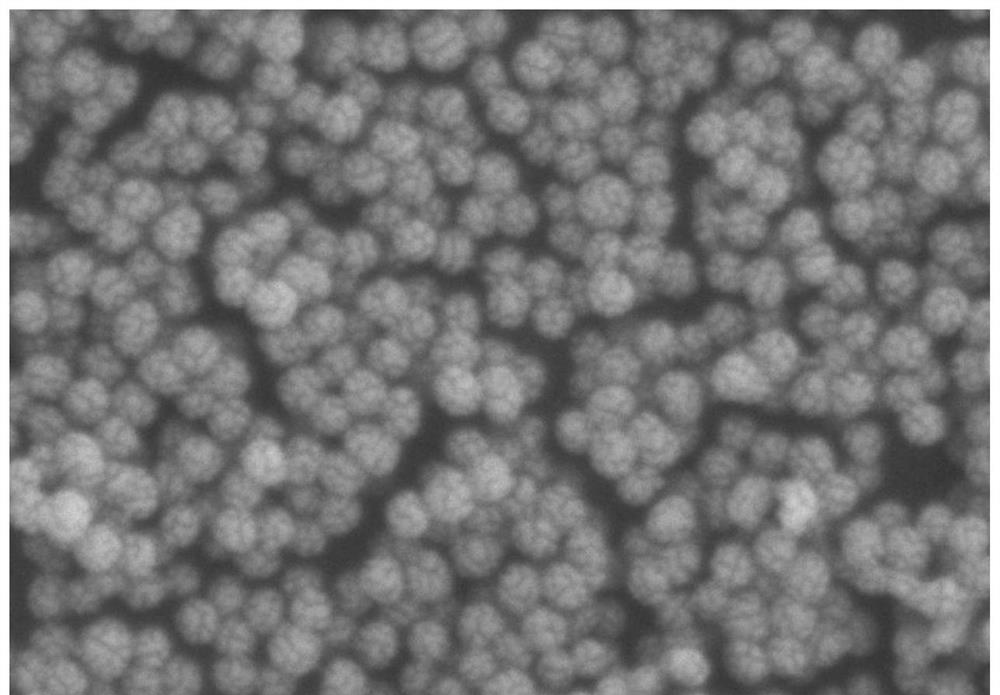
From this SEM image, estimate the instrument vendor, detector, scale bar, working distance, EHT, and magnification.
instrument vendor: Hitachi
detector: SE(L)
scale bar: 400 nm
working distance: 15.9 mm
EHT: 3 kV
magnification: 120 K X